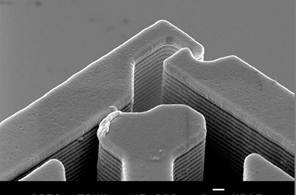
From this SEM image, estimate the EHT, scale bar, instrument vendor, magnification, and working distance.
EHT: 30 kV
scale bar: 1000 nm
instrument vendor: JEOL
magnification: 5 K X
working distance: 15 mm